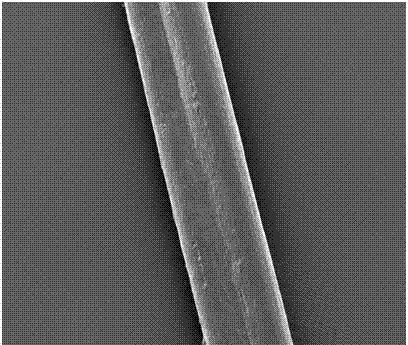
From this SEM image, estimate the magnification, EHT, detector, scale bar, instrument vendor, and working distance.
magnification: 0.3 K X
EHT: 5 kV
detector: ETD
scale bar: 200000 nm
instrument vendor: FEI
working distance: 11.6 mm